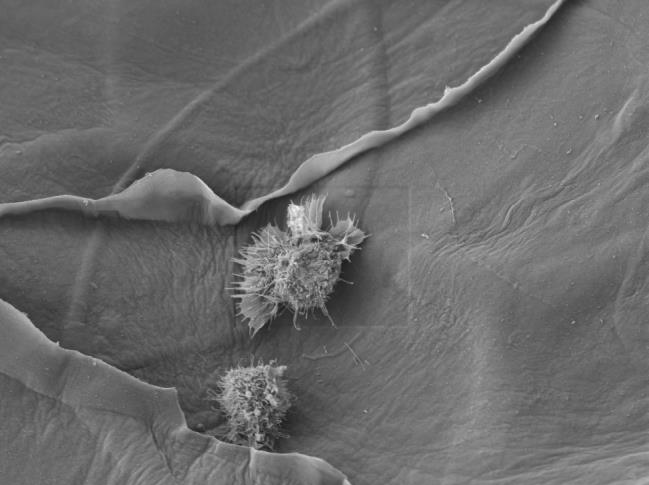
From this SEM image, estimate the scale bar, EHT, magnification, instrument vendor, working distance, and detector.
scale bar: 10000 nm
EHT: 5 kV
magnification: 1 K X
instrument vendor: JEOL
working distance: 10.3 mm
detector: LEI